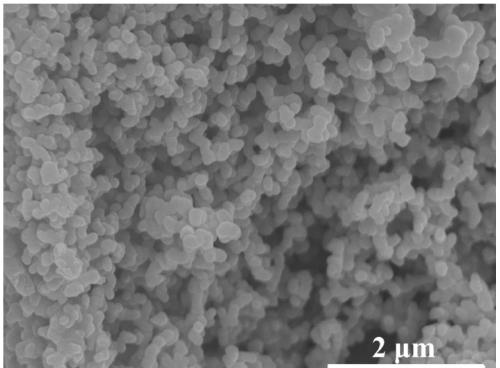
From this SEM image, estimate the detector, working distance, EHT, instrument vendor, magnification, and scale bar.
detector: SE(U)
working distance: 8.7 mm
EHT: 10 kV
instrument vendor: Hitachi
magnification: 20 K X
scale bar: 2000 nm